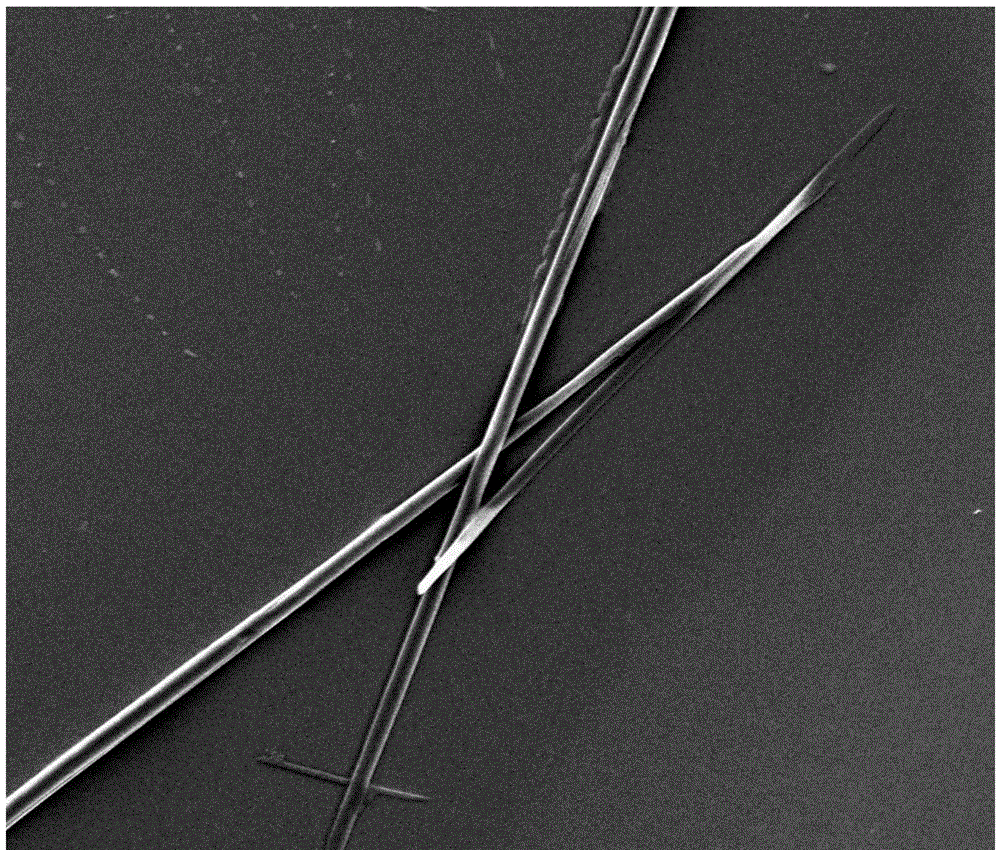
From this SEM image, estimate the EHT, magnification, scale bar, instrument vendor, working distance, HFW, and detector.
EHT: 10 kV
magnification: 2 K X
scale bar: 50000 nm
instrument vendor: FEI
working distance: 9.8 mm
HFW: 149 µm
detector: ETD